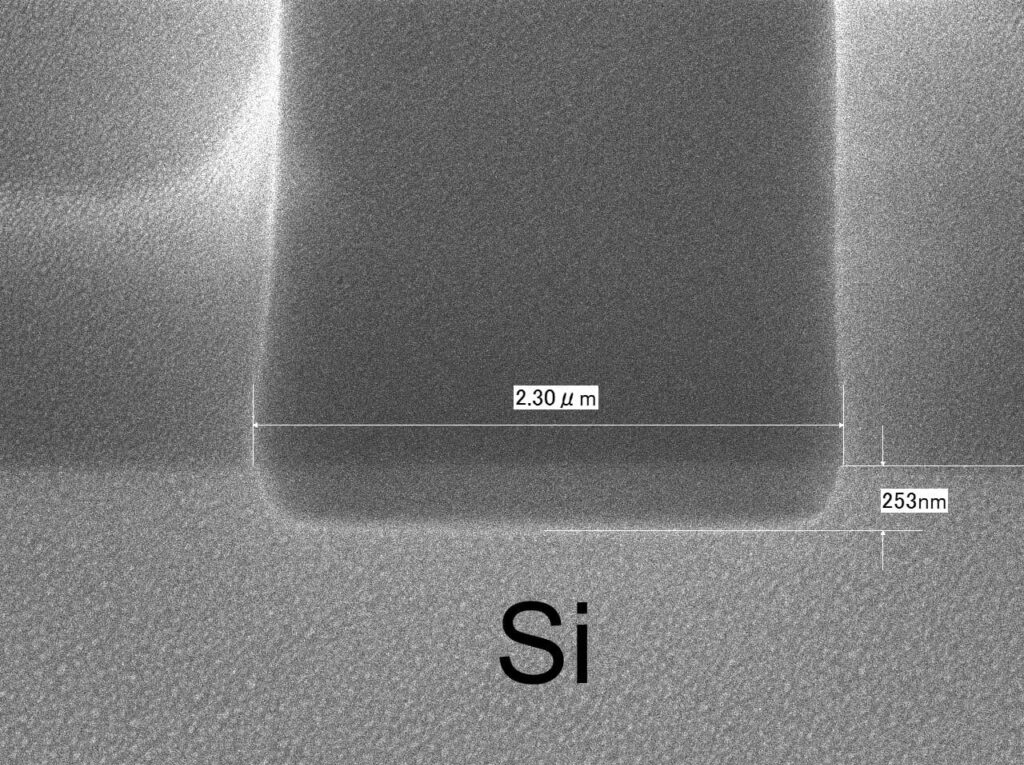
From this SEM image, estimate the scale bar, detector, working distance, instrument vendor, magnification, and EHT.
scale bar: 100 nm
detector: SEI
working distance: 6.3 mm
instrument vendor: JEOL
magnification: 30 K X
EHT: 10 kV